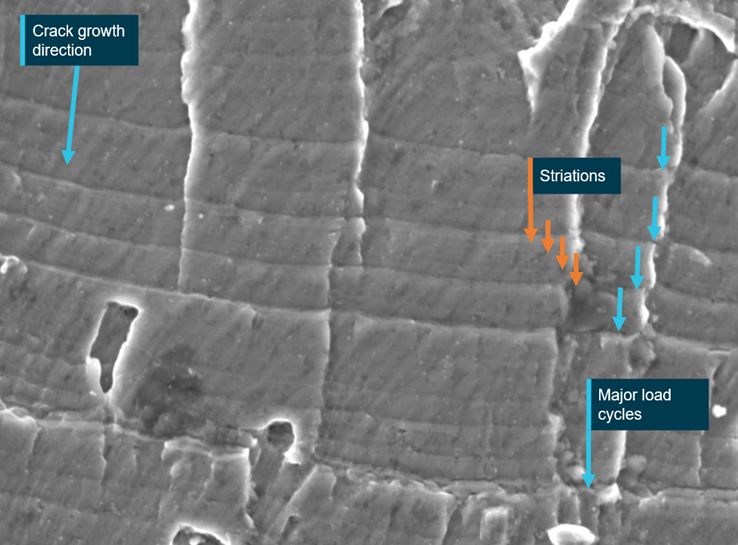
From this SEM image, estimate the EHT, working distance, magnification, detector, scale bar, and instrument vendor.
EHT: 15 kV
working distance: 13.4 mm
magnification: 1.6 K X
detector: SED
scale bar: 10000 nm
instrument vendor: other